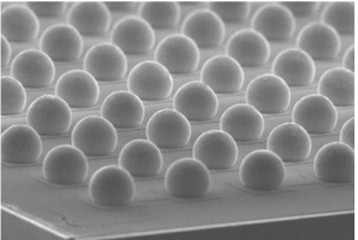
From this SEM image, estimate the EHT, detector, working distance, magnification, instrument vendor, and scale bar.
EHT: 15 kV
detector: SE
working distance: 21.8 mm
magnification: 0.12 K X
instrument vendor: JEOL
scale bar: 250000 nm